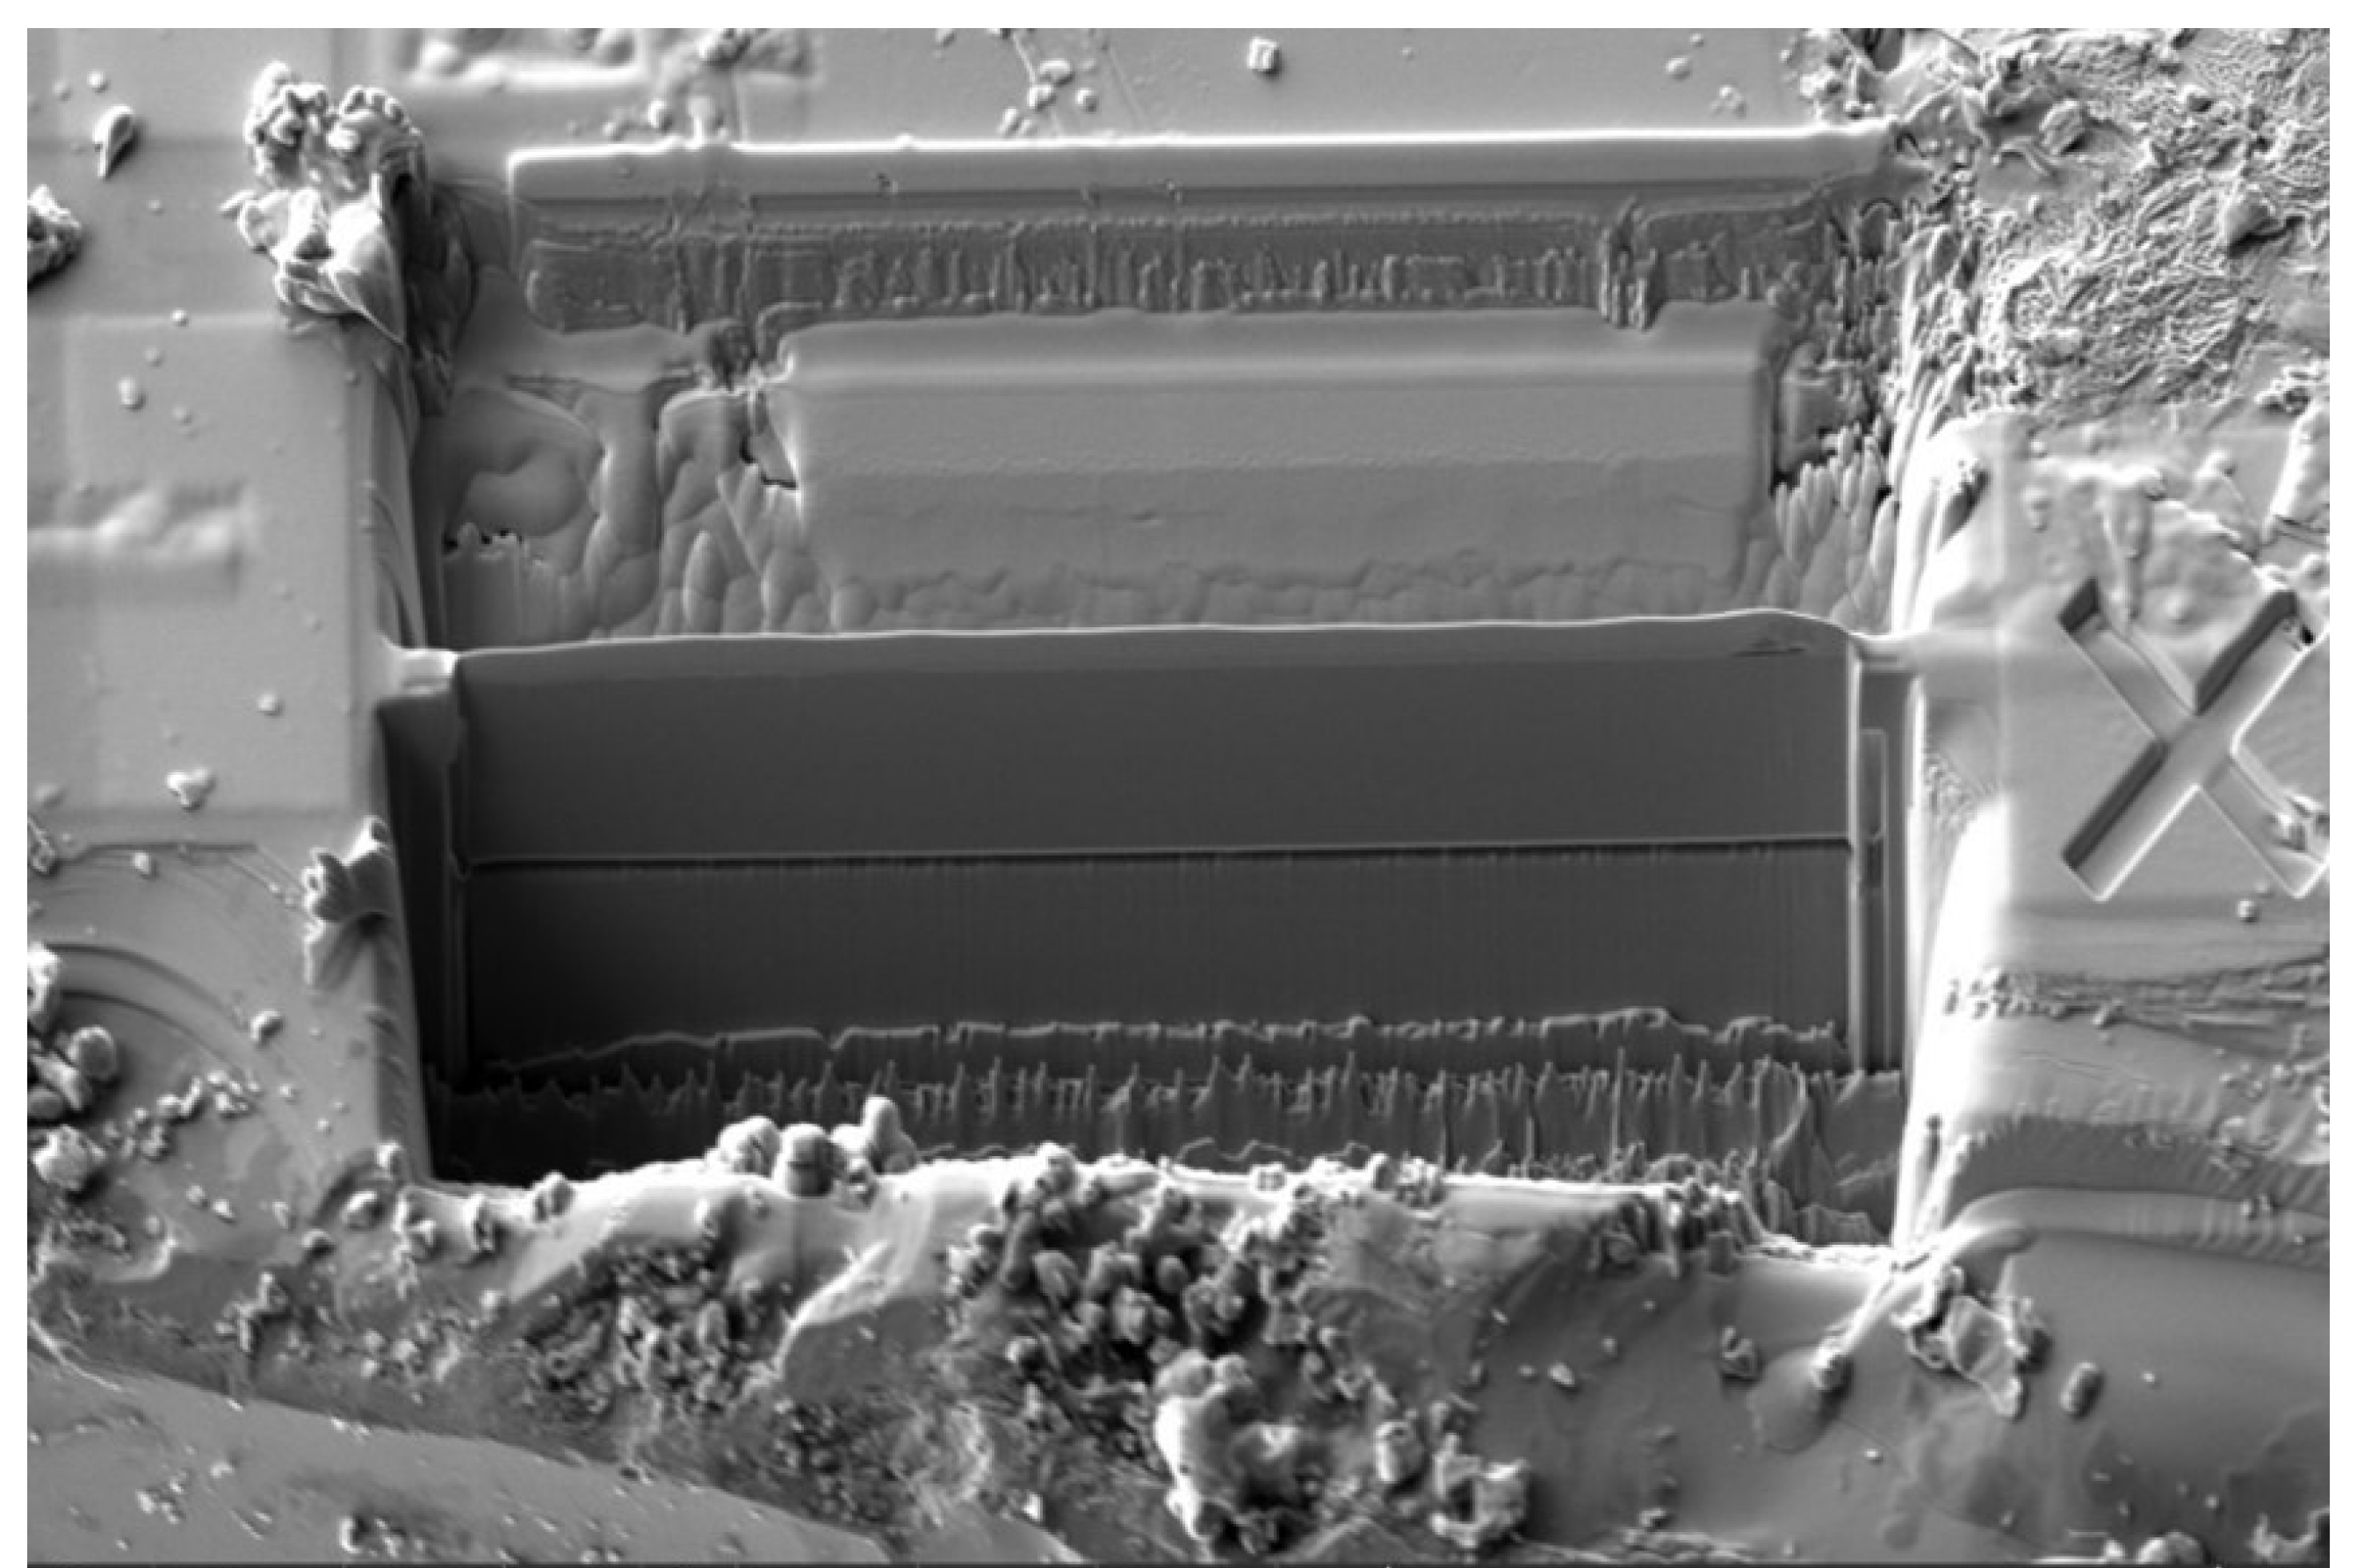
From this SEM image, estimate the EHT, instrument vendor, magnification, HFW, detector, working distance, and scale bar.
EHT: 5 kV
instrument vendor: FEI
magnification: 2.5 K X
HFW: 82.9 µm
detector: ICE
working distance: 7 mm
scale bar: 30000 nm